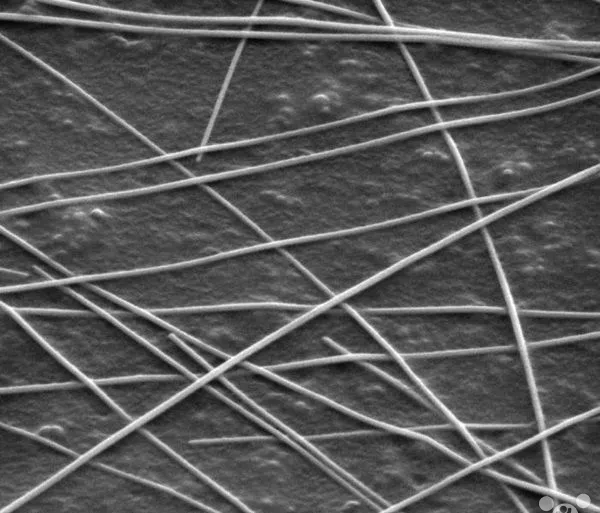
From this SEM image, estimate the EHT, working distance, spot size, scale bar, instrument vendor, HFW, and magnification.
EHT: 5 kV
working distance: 7.5 mm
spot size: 4.5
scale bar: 1000 nm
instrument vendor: FEI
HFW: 2.98 µm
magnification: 50 K X